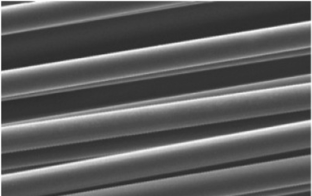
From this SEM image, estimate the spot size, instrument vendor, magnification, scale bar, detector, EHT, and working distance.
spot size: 2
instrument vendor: FEI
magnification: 1.6 K X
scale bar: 20000 nm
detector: SE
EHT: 15 kV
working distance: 10.4 mm